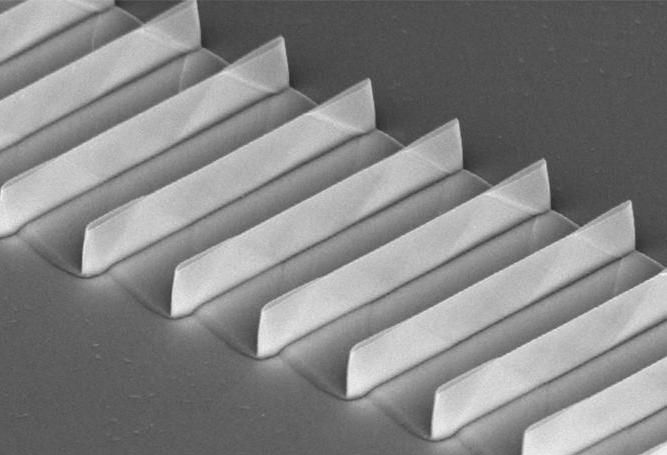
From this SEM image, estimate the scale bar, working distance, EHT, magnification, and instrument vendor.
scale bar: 3000 nm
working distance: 14.1 mm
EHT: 5 kV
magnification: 15 K X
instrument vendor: Hitachi